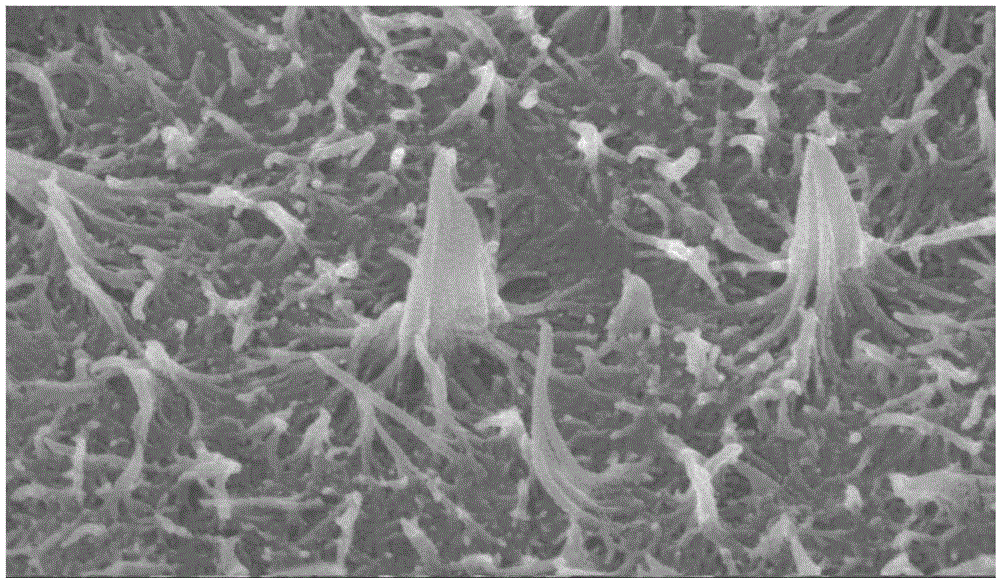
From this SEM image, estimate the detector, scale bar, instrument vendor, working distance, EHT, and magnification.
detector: SEI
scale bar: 100 nm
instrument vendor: JEOL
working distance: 8.2 mm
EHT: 10 kV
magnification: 30 K X